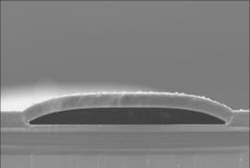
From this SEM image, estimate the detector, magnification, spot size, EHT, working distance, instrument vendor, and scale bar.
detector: SE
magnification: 2 K X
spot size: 2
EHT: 10 kV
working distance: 10 mm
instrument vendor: FEI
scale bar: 10000 nm